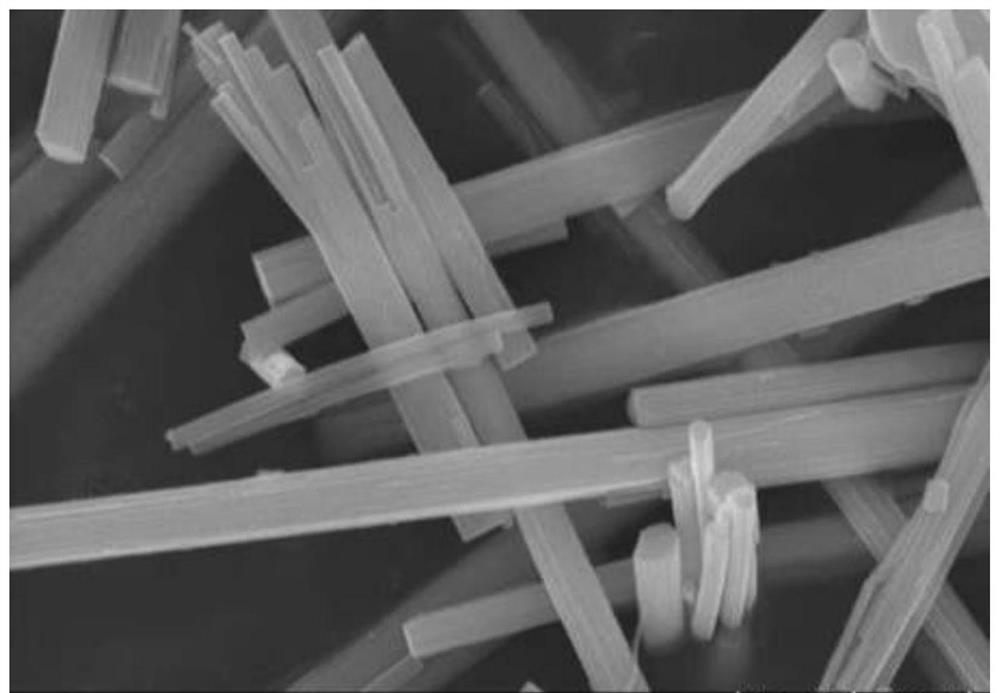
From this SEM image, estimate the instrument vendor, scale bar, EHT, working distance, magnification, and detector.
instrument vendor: Hitachi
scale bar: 1000 nm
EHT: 15 kV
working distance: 10.7 mm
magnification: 30 K X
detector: SE(U)